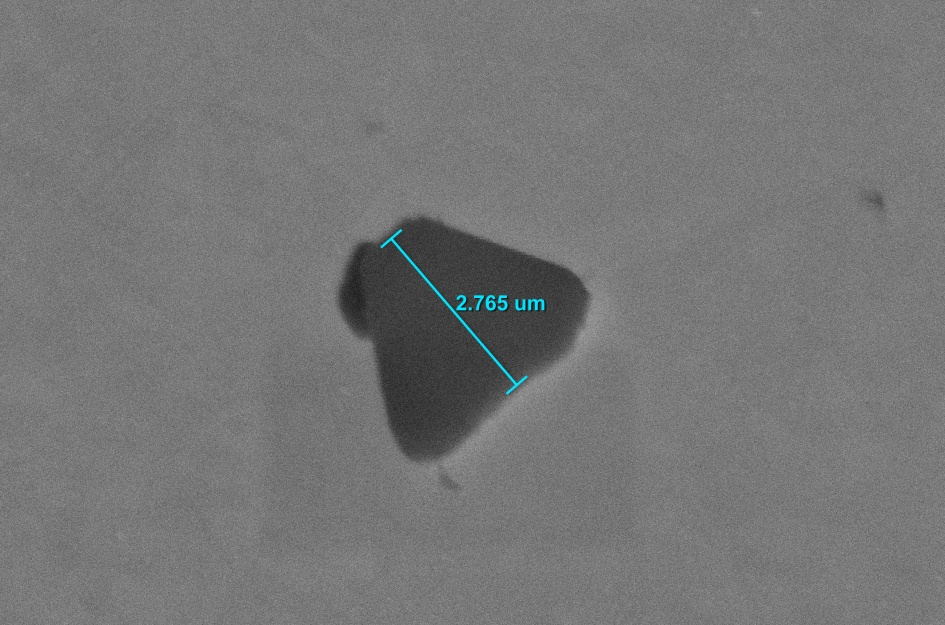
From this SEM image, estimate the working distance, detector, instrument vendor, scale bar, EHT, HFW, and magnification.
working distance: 8 mm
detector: ETD SE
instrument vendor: other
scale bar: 1000 nm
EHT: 15 kV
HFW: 13.55 µm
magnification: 30 K X